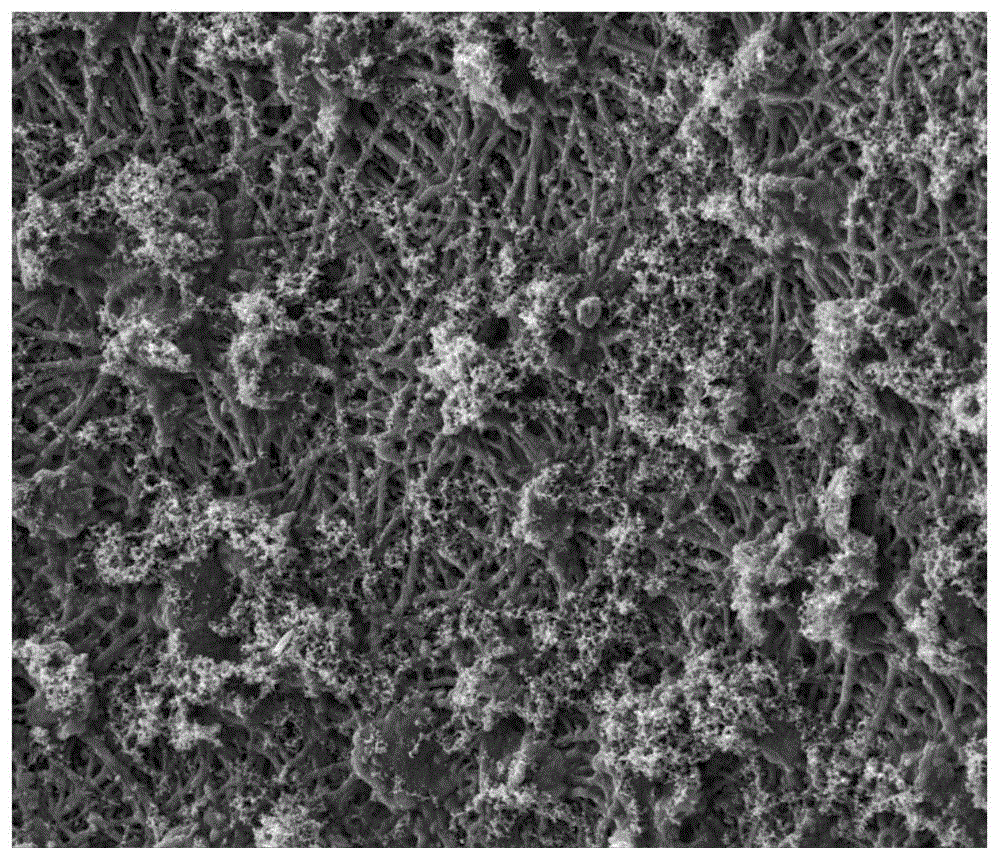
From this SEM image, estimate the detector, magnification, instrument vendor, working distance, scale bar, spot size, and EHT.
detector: SE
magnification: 1 K X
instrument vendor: FEI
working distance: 6.3 mm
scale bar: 100000 nm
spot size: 3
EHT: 15 kV